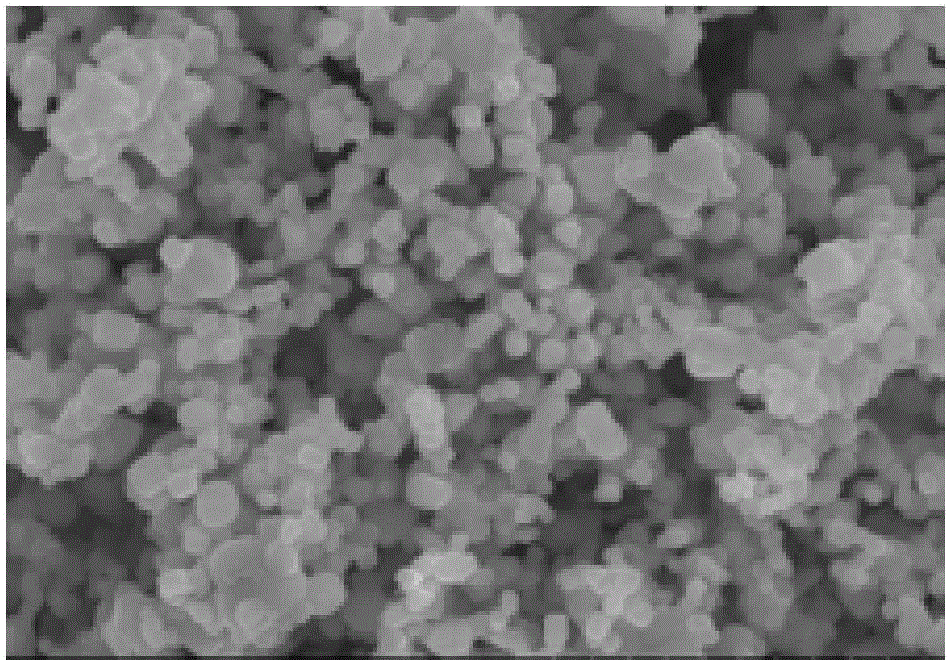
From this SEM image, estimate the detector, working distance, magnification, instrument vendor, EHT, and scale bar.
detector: SE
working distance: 9.5 mm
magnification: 10 K X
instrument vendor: Hitachi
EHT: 15 kV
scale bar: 5000 nm